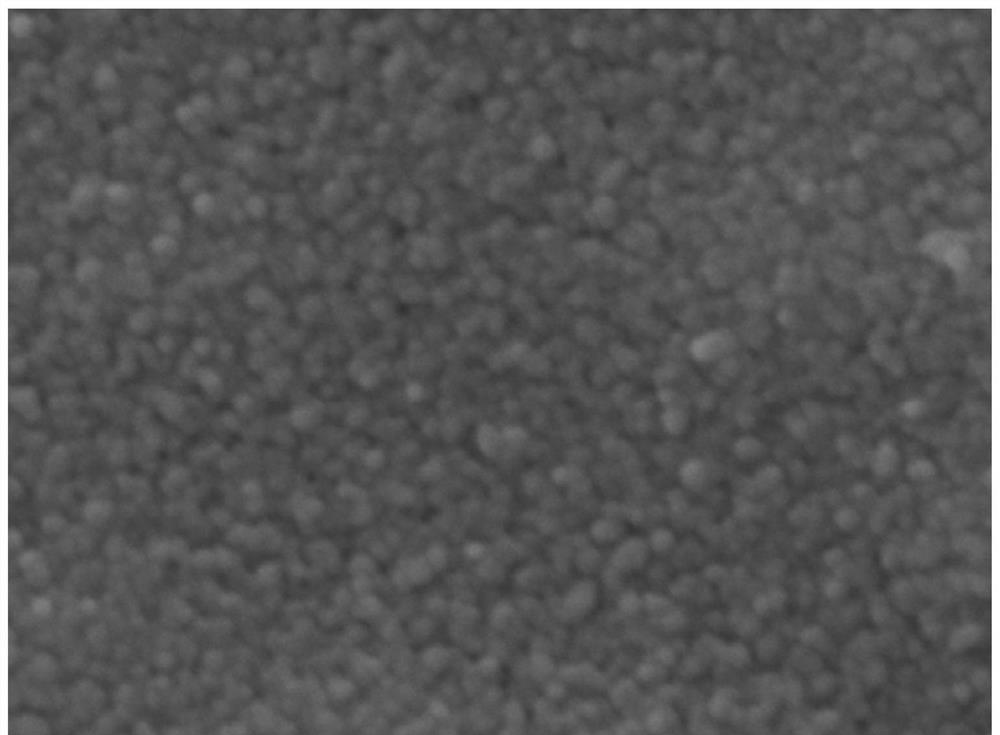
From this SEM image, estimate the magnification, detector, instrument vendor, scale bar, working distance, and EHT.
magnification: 100 K X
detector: LED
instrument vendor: JEOL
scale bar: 100 nm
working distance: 10.1 mm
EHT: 10 kV